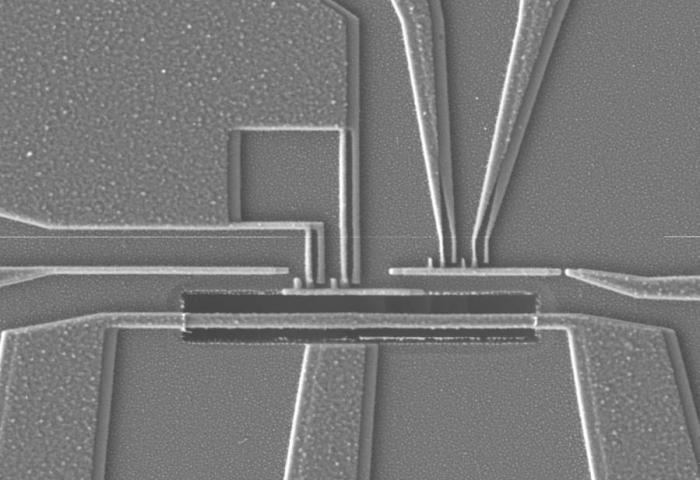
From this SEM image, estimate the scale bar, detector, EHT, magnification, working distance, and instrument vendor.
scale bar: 1000 nm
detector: LEI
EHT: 3 kV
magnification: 10 K X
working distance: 8 mm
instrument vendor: JEOL